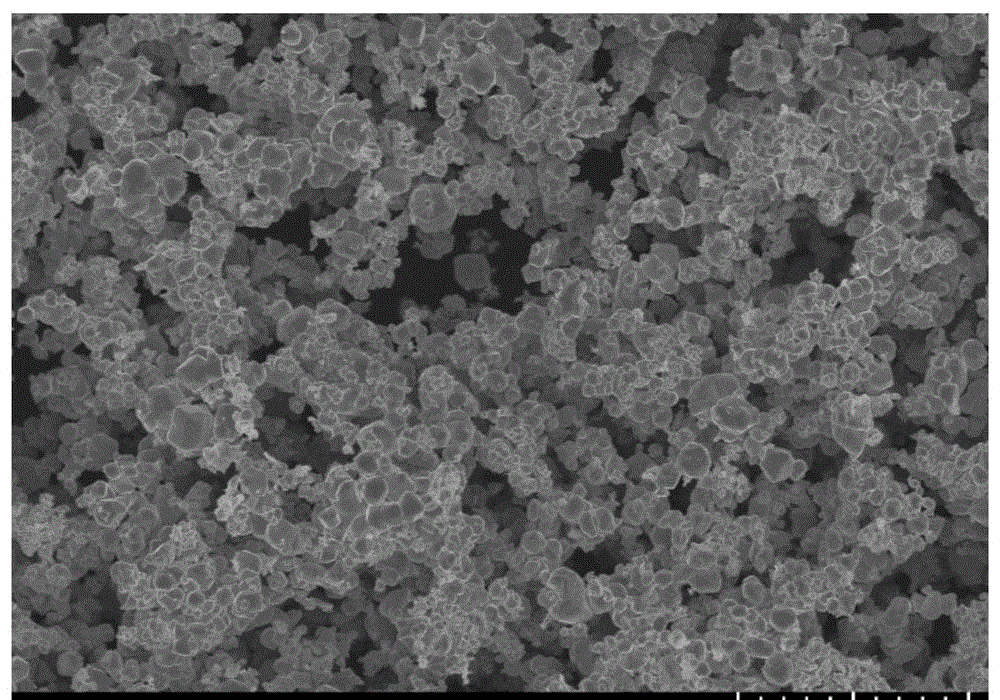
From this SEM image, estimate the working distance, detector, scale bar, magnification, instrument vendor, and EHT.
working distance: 11.1 mm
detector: SE(U)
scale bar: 10000 nm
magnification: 3 K X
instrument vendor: Hitachi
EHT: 10 kV